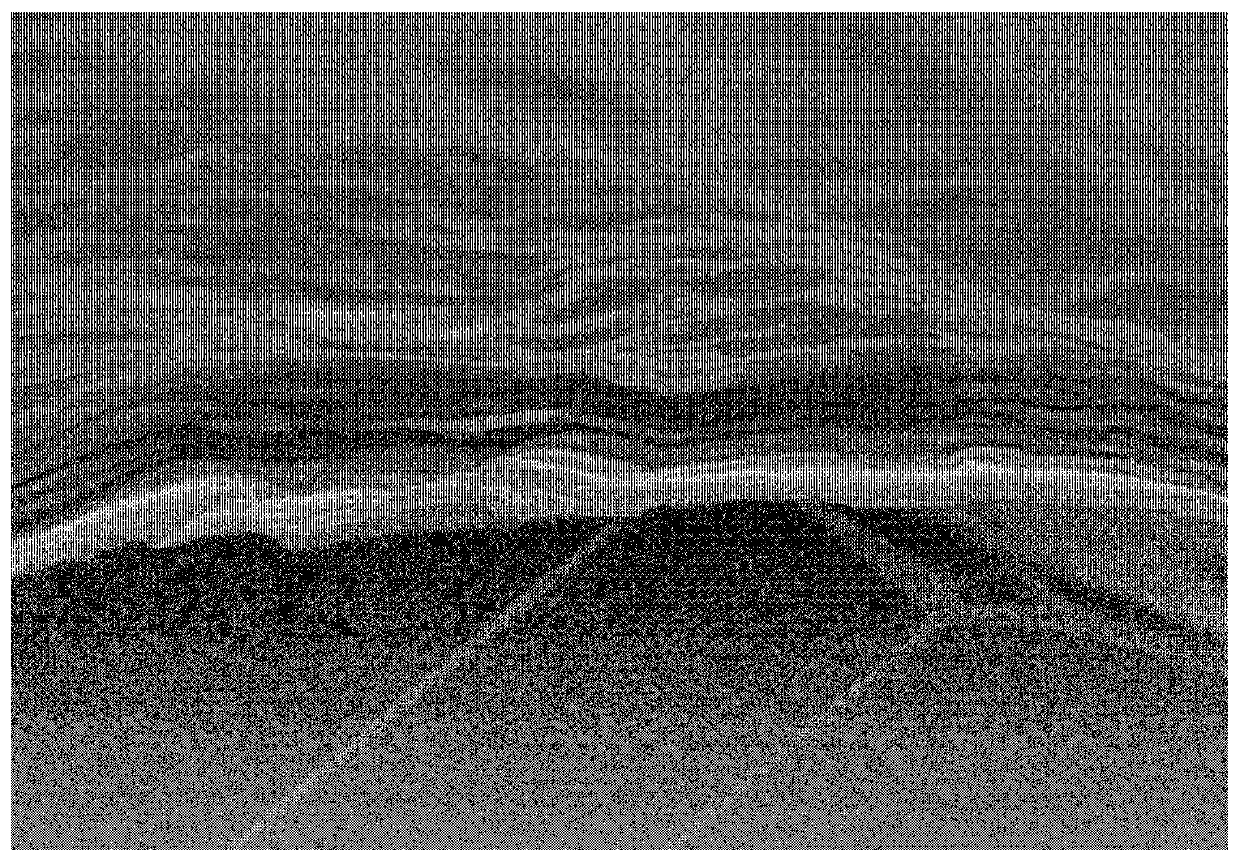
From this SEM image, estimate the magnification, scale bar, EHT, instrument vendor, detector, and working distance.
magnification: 50 K X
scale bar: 1000 nm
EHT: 3 kV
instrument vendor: Hitachi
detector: SE(U)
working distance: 9.1 mm